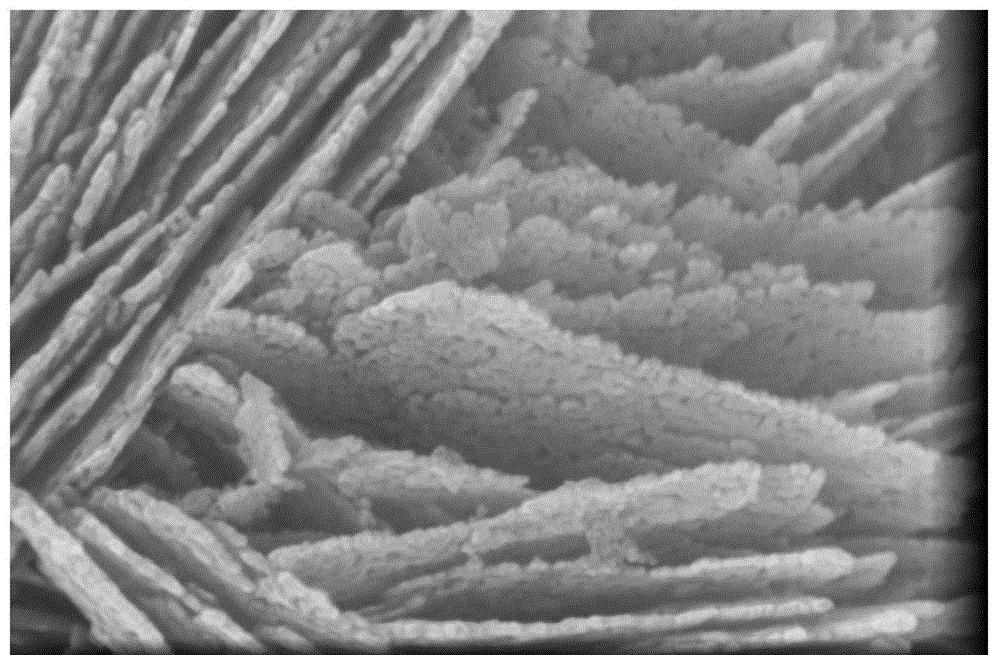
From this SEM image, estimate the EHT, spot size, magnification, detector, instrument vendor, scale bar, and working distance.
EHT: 15 kV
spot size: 3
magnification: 350 K X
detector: TLD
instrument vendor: FEI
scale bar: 400 nm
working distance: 4.8 mm